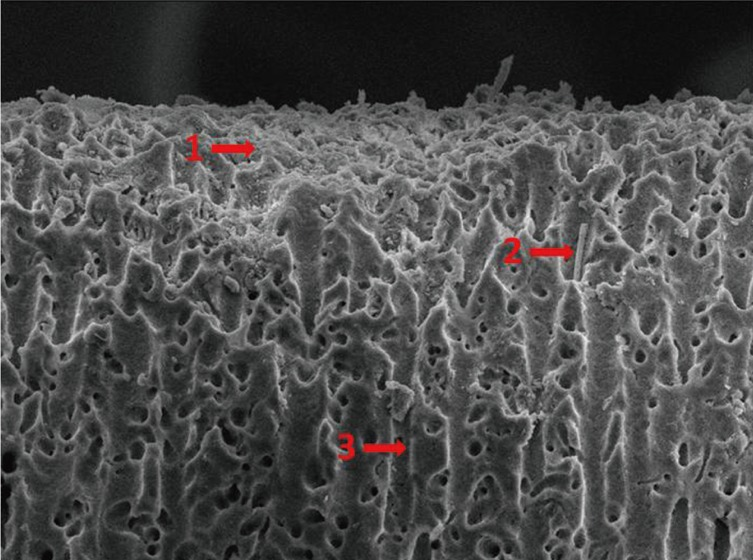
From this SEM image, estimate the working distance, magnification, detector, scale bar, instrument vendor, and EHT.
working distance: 9.7 mm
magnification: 1 K X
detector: SEI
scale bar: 10000 nm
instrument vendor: JEOL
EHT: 10 kV